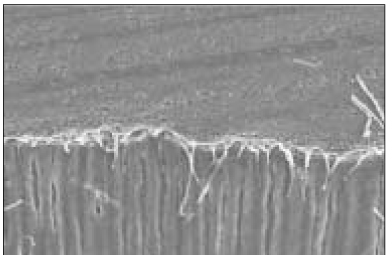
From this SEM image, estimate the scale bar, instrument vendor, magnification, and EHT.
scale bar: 50000 nm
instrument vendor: JEOL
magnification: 0.5 K X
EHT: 15 kV